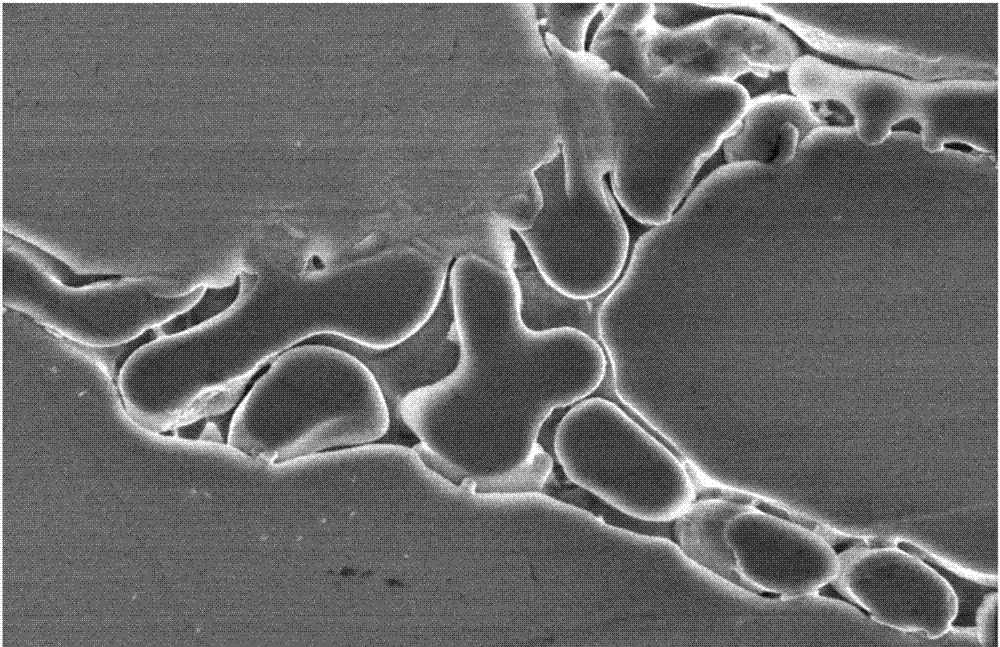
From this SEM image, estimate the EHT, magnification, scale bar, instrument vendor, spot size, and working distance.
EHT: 2 kV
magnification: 8 K X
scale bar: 10000 nm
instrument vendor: other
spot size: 3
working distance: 7.9 mm